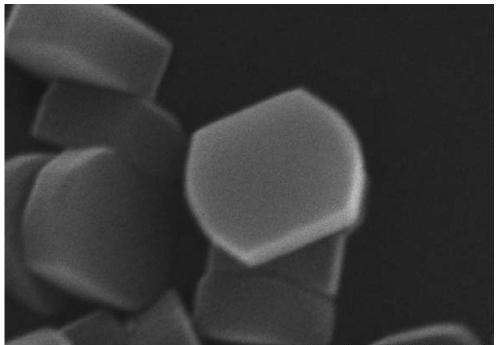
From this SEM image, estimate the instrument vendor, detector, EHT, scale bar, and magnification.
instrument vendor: Hitachi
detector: SE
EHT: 15 kV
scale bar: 1000 nm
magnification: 50 K X